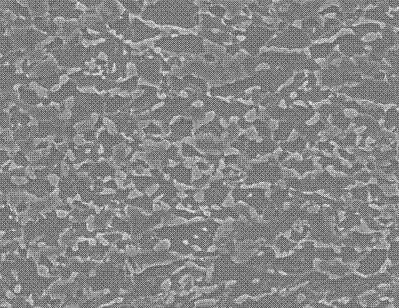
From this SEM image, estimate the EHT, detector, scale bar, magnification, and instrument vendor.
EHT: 25 kV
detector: SEI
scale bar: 10000 nm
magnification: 1.5 K X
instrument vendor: JEOL